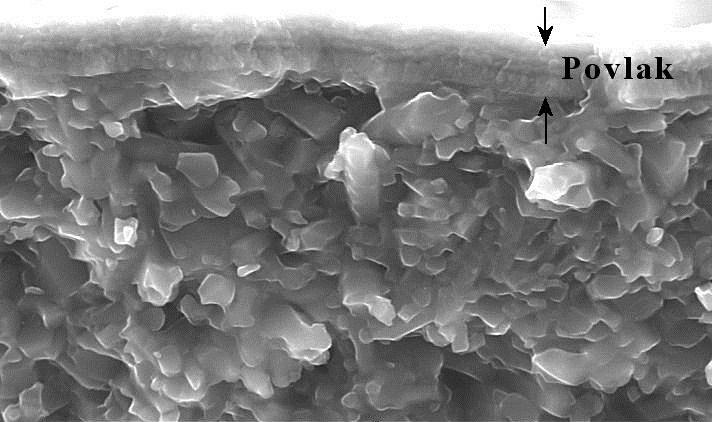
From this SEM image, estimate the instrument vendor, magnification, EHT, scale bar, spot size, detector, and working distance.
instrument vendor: FEI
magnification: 4 K X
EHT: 20 kV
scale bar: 5000 nm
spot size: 4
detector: SE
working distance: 11.6 mm